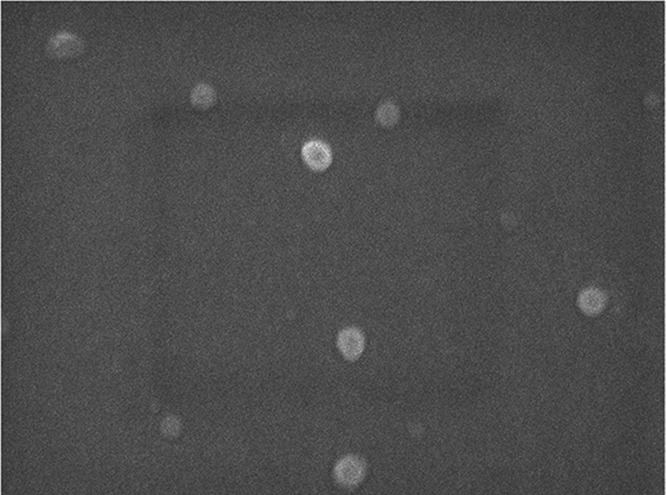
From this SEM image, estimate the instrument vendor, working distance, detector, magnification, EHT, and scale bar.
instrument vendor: JEOL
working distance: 7.6 mm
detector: SEI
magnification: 40 K X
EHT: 3 kV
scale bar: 100 nm